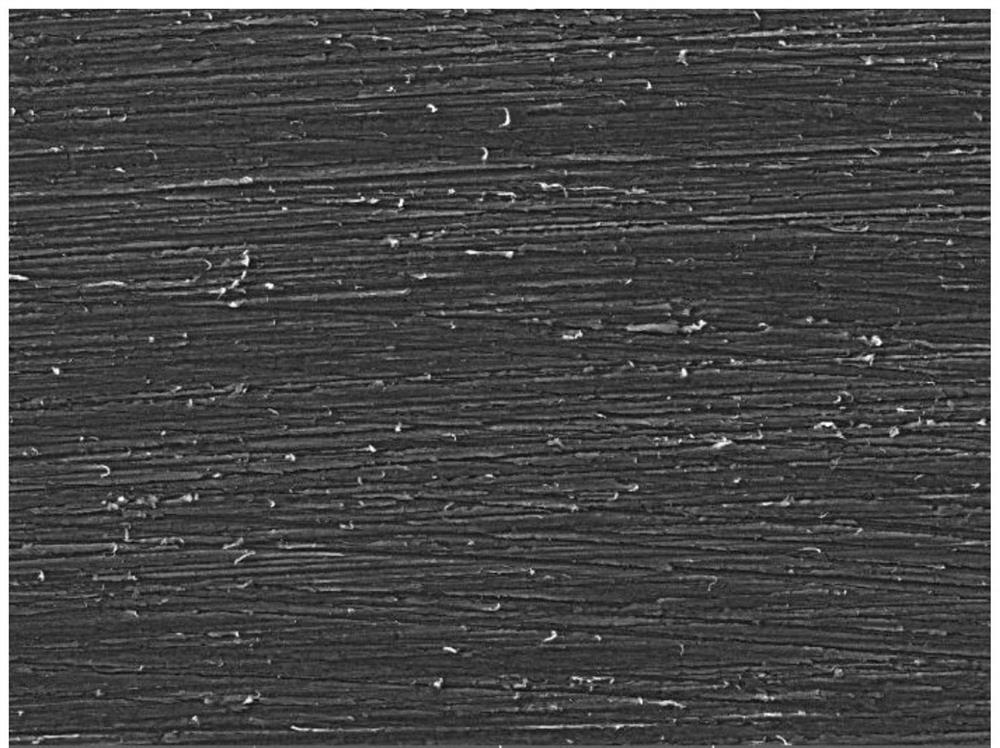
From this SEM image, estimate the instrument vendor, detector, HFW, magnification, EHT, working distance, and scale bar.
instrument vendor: Tescan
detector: SE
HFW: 923 µm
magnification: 0.3 K X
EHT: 20 kV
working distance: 25.65 mm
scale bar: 200000 nm